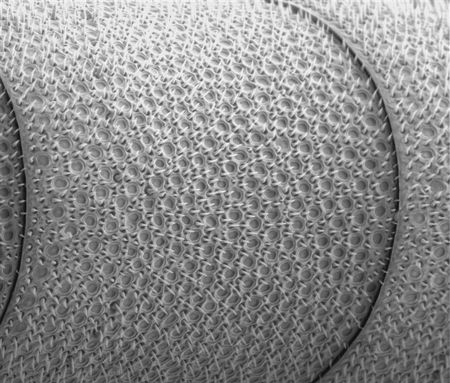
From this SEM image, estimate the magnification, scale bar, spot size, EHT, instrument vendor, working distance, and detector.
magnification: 0.7 K X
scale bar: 100000 nm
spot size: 4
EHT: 12 kV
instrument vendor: FEI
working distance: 11.5 mm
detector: SE